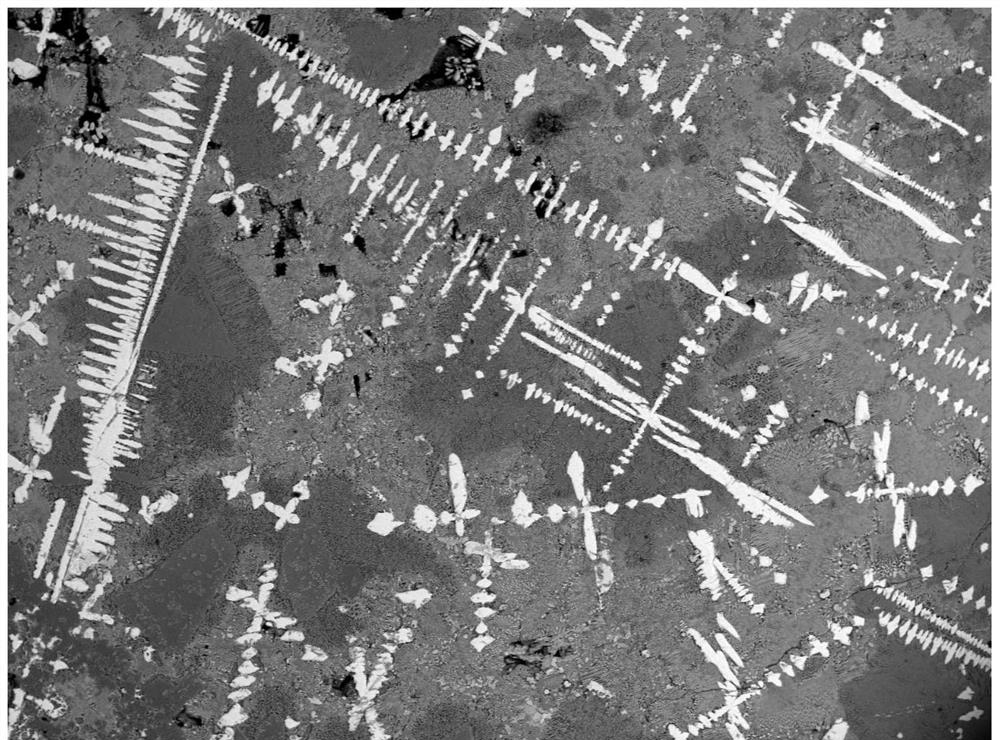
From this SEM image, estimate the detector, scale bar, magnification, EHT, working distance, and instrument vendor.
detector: BED-S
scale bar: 500000 nm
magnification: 0.05 K X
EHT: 20 kV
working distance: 9.7 mm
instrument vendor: other